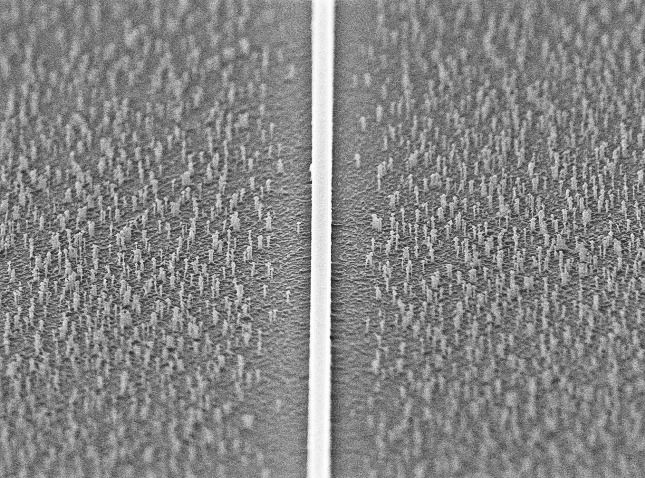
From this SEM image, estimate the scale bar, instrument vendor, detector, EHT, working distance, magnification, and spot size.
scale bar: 2000 nm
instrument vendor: FEI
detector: SE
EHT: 10 kV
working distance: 11.1 mm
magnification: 8.638 K X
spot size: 3